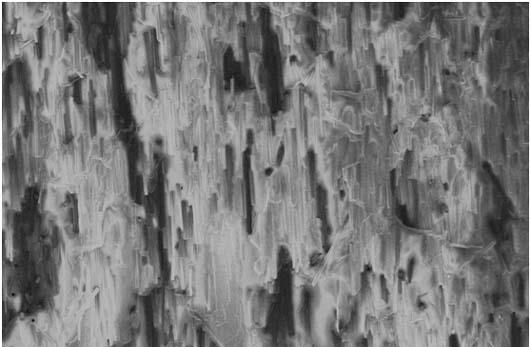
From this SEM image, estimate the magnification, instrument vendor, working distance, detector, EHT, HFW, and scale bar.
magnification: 0.25 K X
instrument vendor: Thermo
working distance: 7.3 mm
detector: ETD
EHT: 3 kV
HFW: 1660 µm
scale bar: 500000 nm